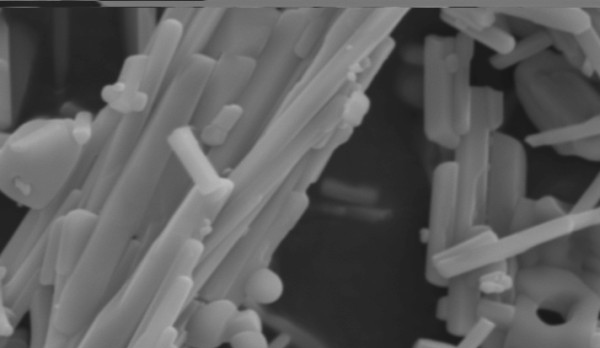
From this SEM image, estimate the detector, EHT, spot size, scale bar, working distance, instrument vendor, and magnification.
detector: SE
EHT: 15 kV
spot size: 5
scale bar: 5000 nm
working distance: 10 mm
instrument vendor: FEI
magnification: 5.146 K X